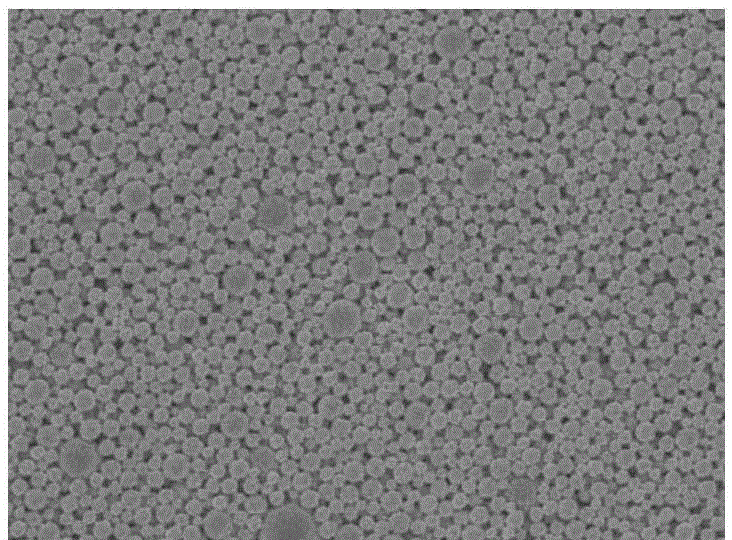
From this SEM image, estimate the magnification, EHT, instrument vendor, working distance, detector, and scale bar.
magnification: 10 K X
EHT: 5 kV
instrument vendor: JEOL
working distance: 6.7 mm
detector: SEI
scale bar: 1000 nm